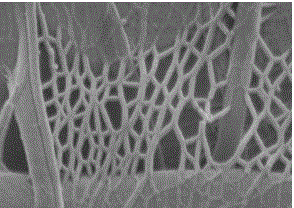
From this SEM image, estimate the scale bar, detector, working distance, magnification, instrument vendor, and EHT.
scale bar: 100 nm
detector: SEI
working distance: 4.3 mm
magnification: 50 K X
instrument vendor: JEOL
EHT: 2 kV